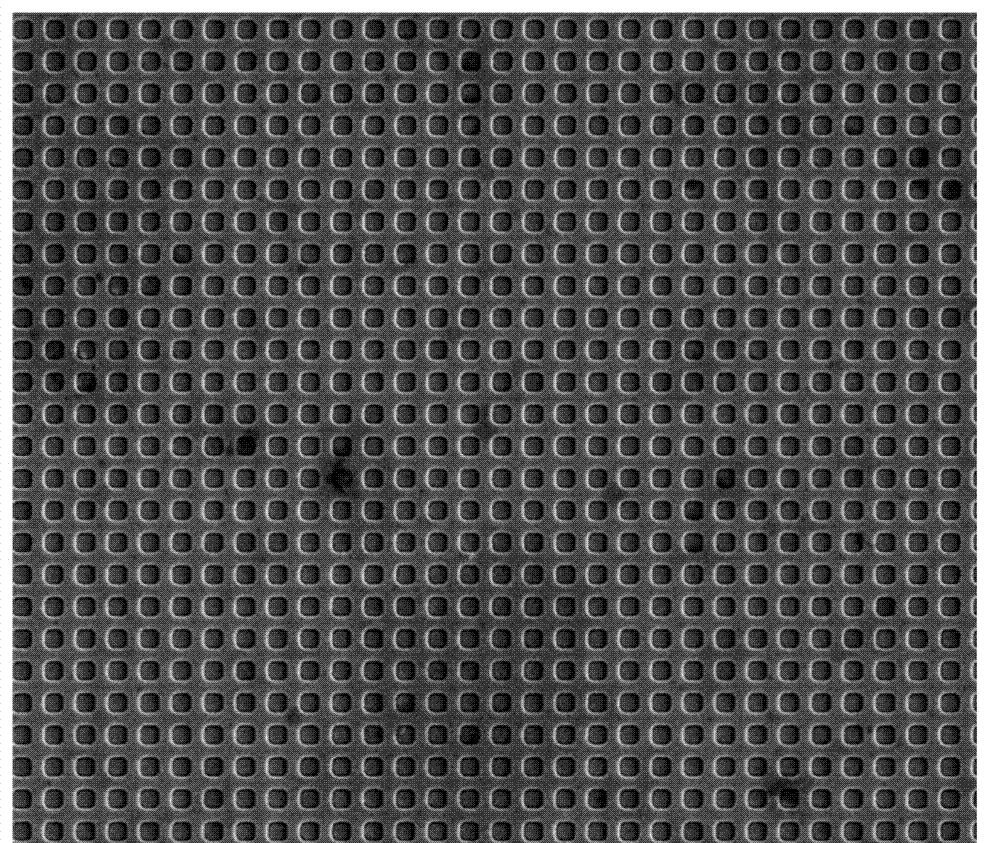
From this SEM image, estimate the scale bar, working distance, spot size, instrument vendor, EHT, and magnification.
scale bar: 10000 nm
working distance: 10.1 mm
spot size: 3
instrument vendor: FEI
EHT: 10 kV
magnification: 10 K X